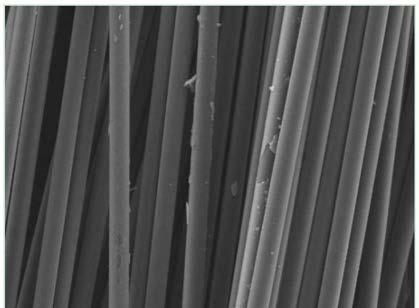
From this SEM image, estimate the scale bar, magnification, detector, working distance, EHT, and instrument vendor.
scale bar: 20000 nm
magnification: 2 K X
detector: SE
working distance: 14.13 mm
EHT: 9 kV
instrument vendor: Tescan